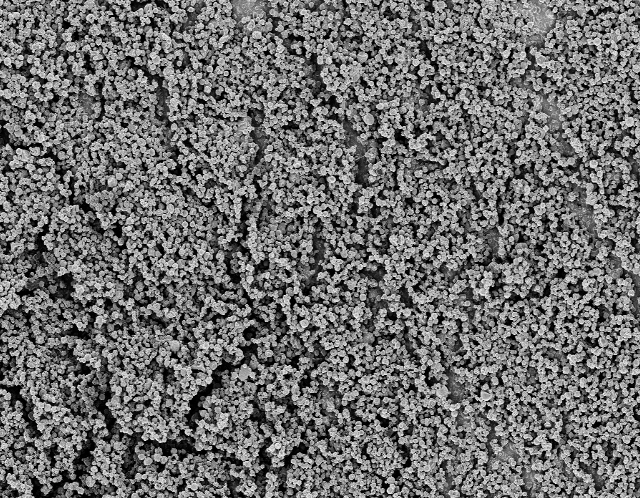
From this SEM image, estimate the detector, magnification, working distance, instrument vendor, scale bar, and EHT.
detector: InLens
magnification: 2 K X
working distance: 4 mm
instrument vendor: Zeiss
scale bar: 10000 nm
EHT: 3 kV